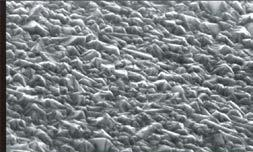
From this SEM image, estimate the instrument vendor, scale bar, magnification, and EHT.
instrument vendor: JEOL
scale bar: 10000 nm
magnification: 2 K X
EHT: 15 kV